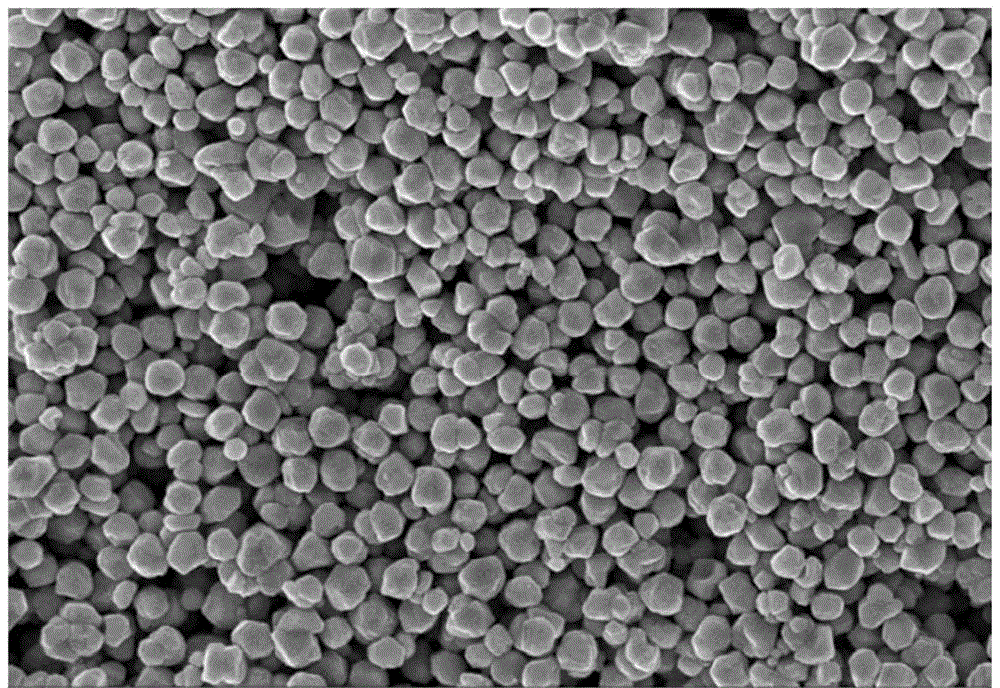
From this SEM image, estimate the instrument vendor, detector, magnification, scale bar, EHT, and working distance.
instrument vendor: Zeiss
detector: InLens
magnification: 5 K X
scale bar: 1000 nm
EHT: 15 kV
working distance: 6.7 mm